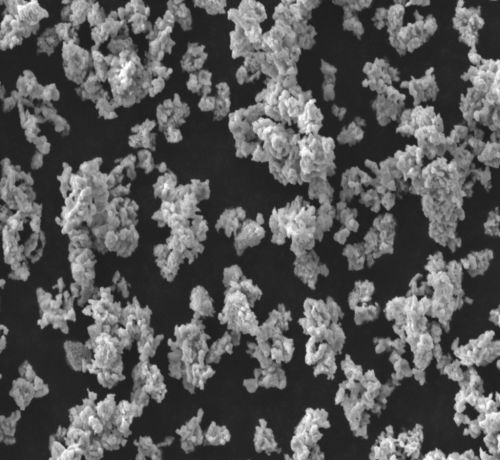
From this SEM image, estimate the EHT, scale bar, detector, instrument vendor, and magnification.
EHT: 15 kV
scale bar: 10000 nm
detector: SEI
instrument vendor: JEOL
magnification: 2 K X